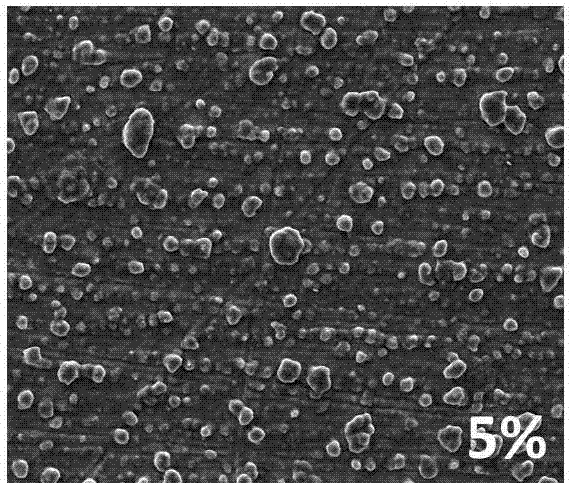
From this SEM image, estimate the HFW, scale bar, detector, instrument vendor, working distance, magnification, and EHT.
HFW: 597 µm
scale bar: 200000 nm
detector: ETD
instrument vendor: FEI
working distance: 13.3 mm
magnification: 0.5 K X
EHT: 20 kV